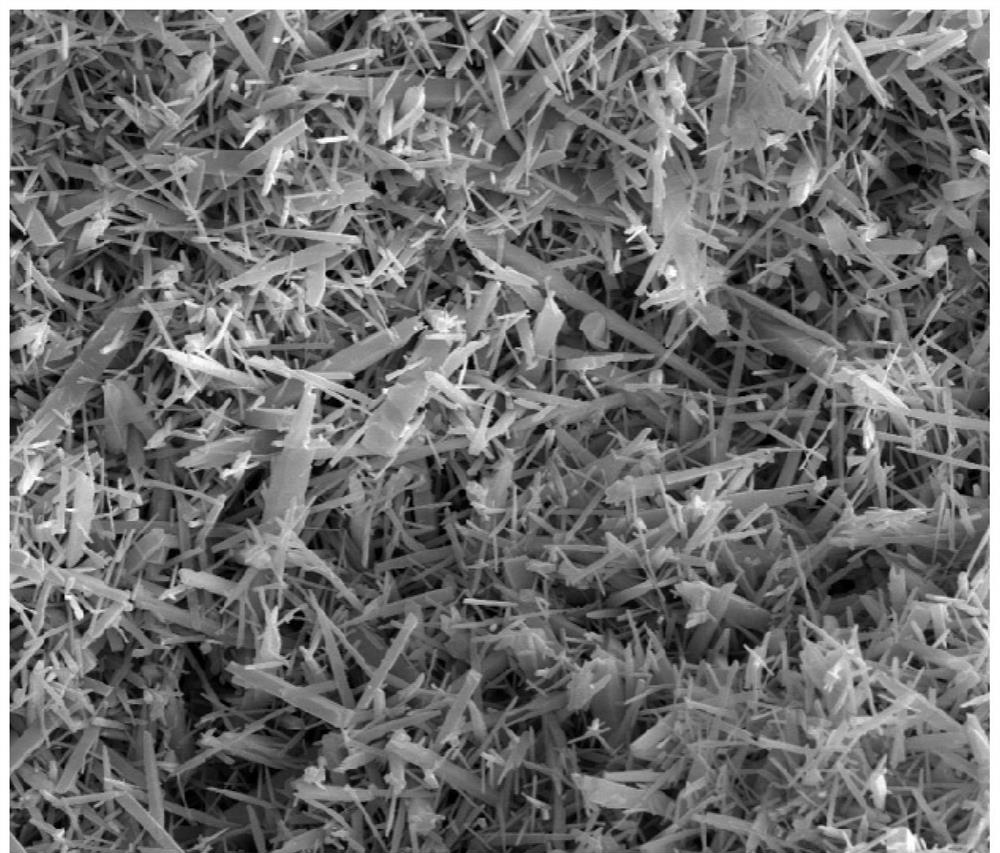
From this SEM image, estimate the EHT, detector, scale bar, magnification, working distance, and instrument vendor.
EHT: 20 kV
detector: SE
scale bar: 10000 nm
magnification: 5 K X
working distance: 5.2 mm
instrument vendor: FEI